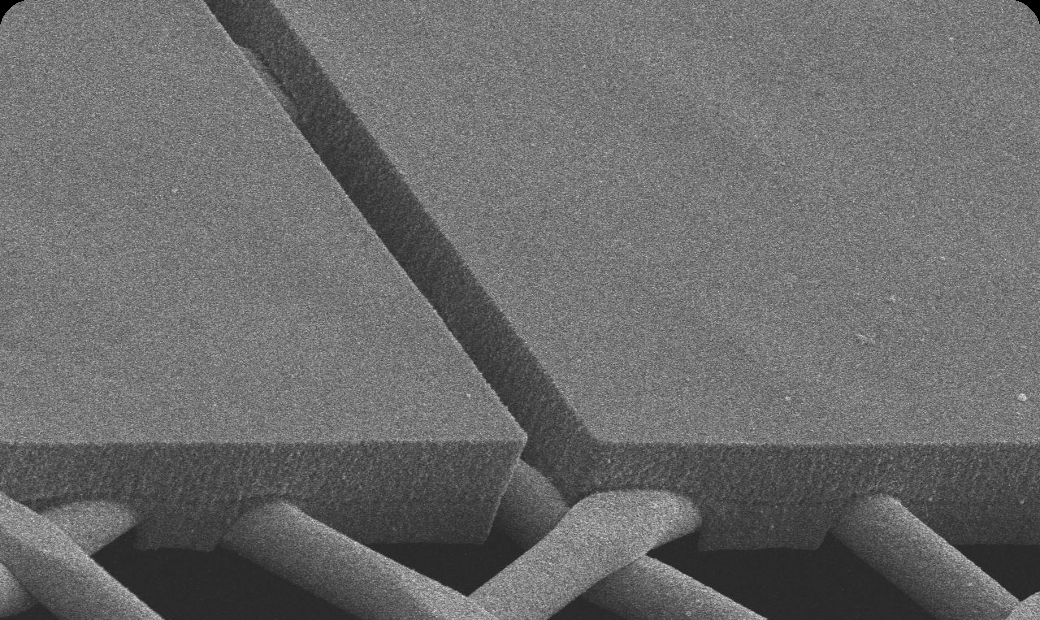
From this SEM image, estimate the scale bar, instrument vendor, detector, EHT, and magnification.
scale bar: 50000 nm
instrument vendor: JEOL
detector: SEI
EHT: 12 kV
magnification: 0.5 K X